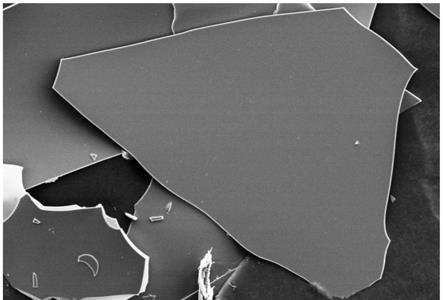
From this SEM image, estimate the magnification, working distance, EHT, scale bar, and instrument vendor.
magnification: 0.2 K X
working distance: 9.2 mm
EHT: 15 kV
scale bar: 200000 nm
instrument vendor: Hitachi